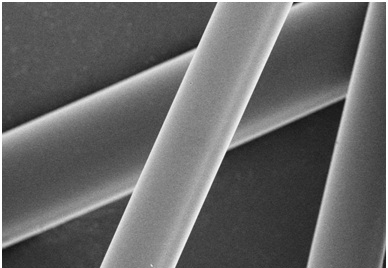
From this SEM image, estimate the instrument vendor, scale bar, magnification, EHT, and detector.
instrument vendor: Hitachi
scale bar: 20000 nm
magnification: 2 K X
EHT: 3 kV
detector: SE(U)